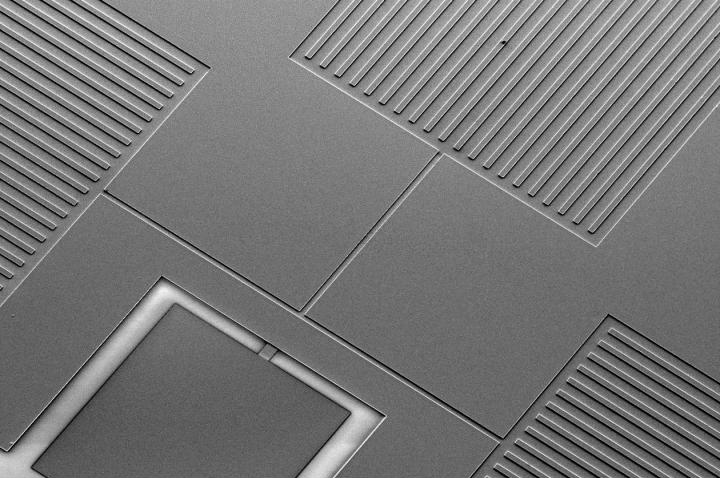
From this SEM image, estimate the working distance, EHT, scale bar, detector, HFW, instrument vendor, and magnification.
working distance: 9.6 mm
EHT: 5 kV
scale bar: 400000 nm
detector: ETD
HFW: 1170 µm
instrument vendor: FEI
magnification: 0.177 K X